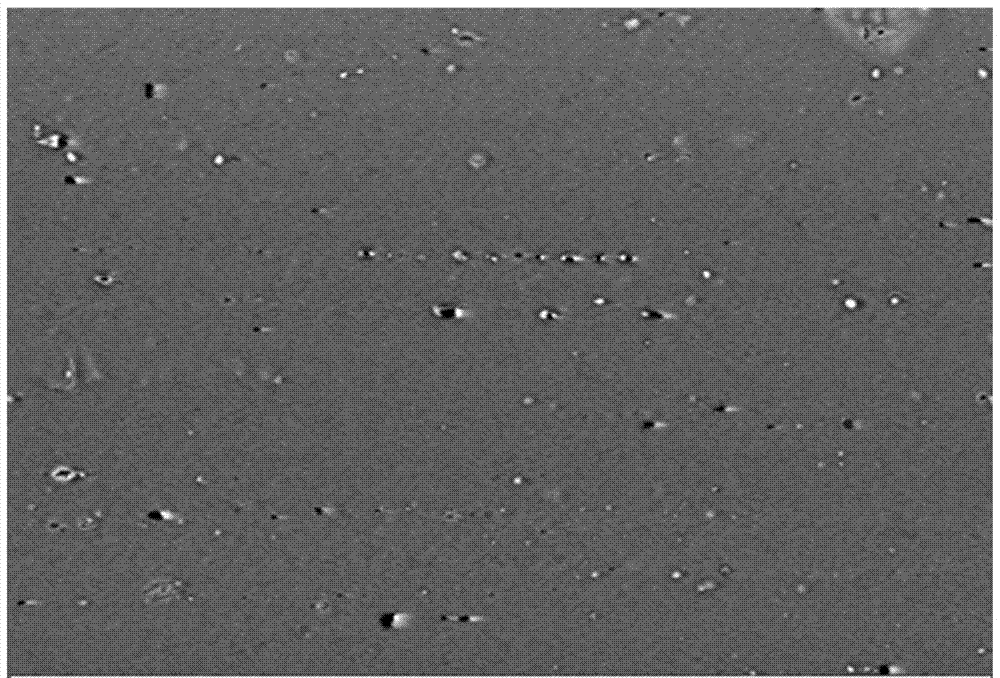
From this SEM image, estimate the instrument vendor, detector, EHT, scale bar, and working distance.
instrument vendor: Zeiss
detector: QBSD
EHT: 20 kV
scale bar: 20000 nm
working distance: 9 mm